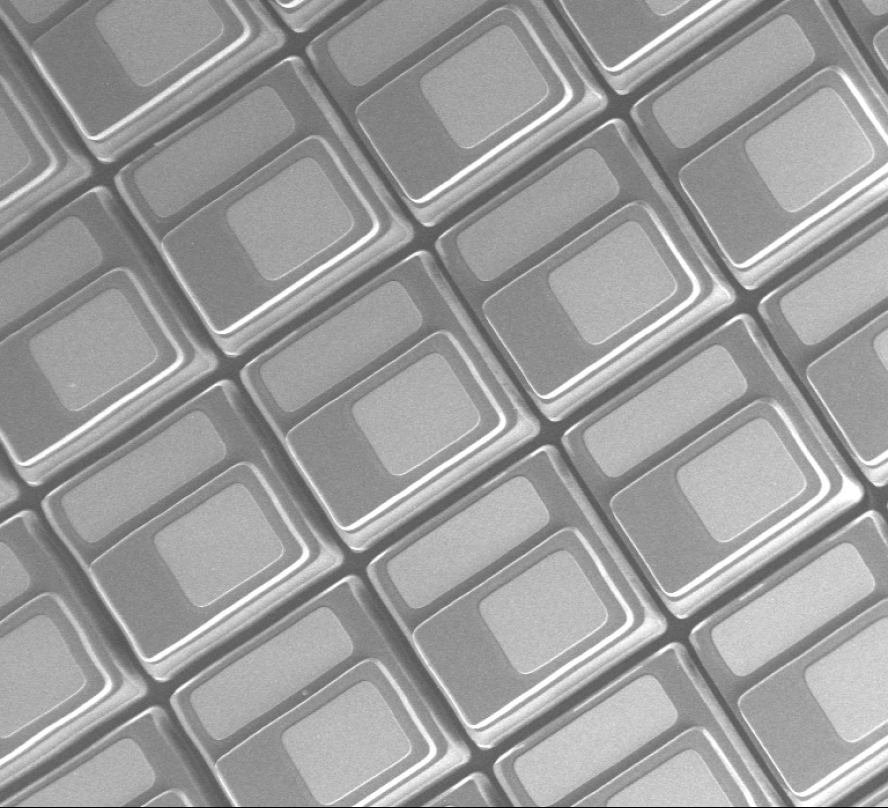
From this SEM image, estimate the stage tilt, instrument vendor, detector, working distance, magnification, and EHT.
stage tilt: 45°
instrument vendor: Zeiss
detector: SE1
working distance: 19.1 mm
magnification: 0.741 K X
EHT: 20 kV